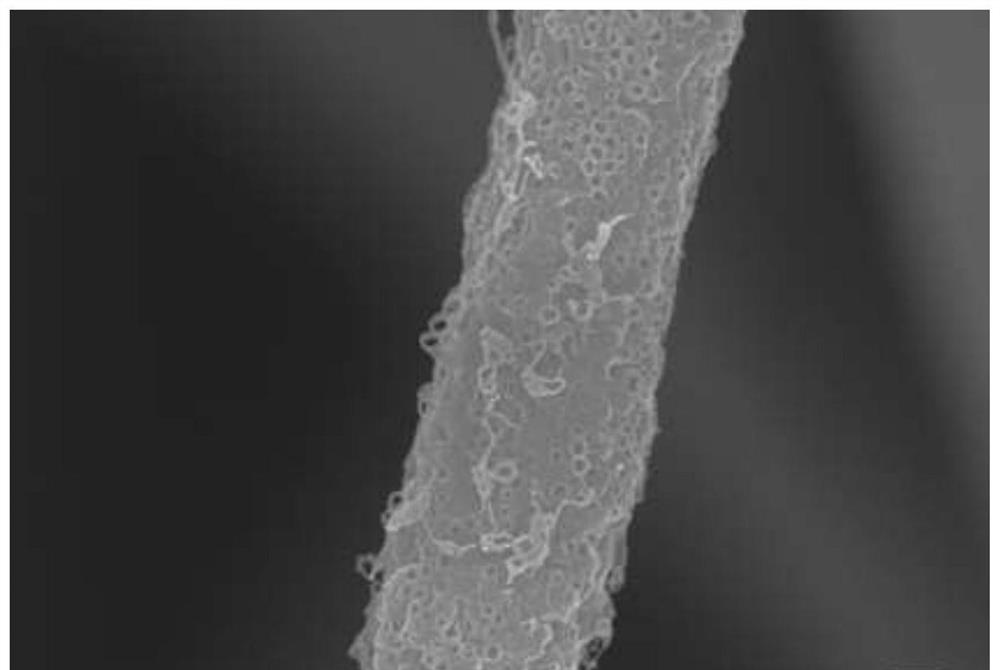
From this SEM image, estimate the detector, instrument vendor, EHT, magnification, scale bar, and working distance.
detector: InLens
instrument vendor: Zeiss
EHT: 10 kV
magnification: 30 K X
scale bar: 300 nm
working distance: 8.6 mm